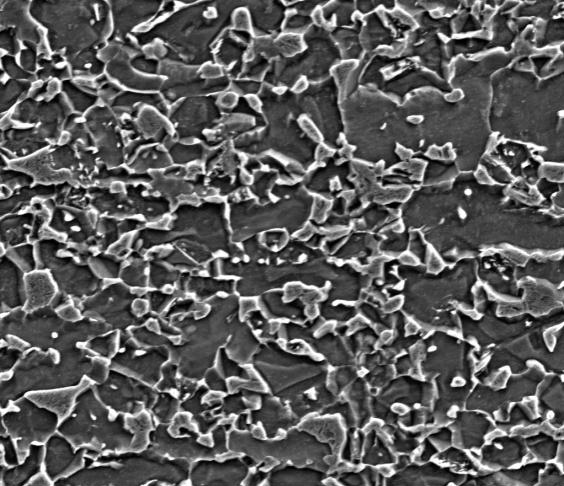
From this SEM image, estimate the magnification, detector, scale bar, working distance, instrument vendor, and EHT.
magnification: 10 K X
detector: ETD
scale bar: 10000 nm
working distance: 7.6 mm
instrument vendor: FEI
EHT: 10 kV